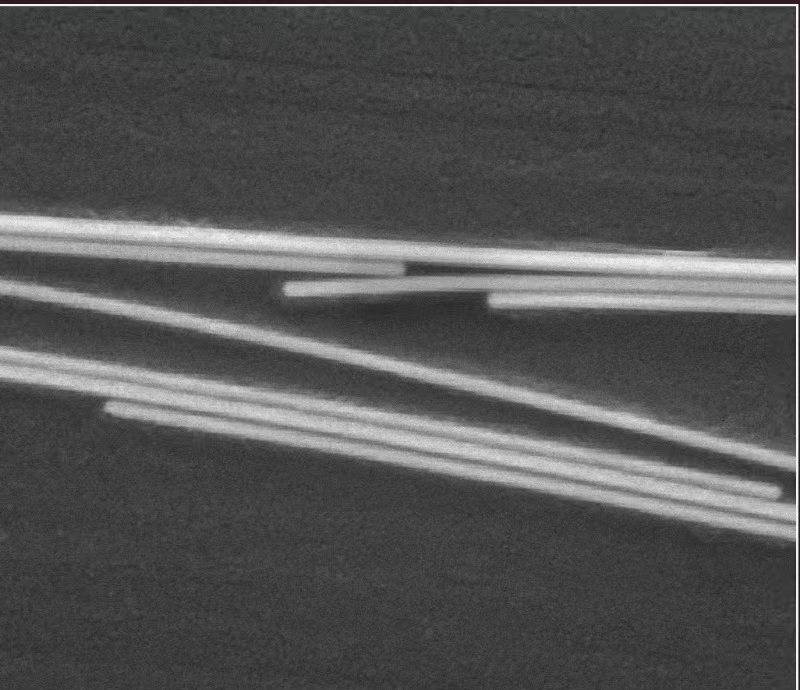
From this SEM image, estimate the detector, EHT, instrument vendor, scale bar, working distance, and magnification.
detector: ETD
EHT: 20 kV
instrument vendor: FEI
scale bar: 1000 nm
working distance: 10.9 mm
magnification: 50 K X